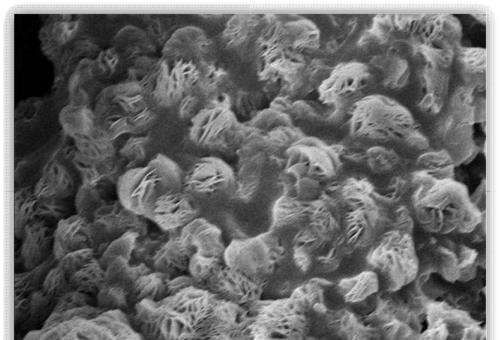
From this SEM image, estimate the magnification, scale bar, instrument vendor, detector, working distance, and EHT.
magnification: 4.5 K X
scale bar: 10000 nm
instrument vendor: Hitachi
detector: SE(U)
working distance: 15.2 mm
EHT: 10 kV